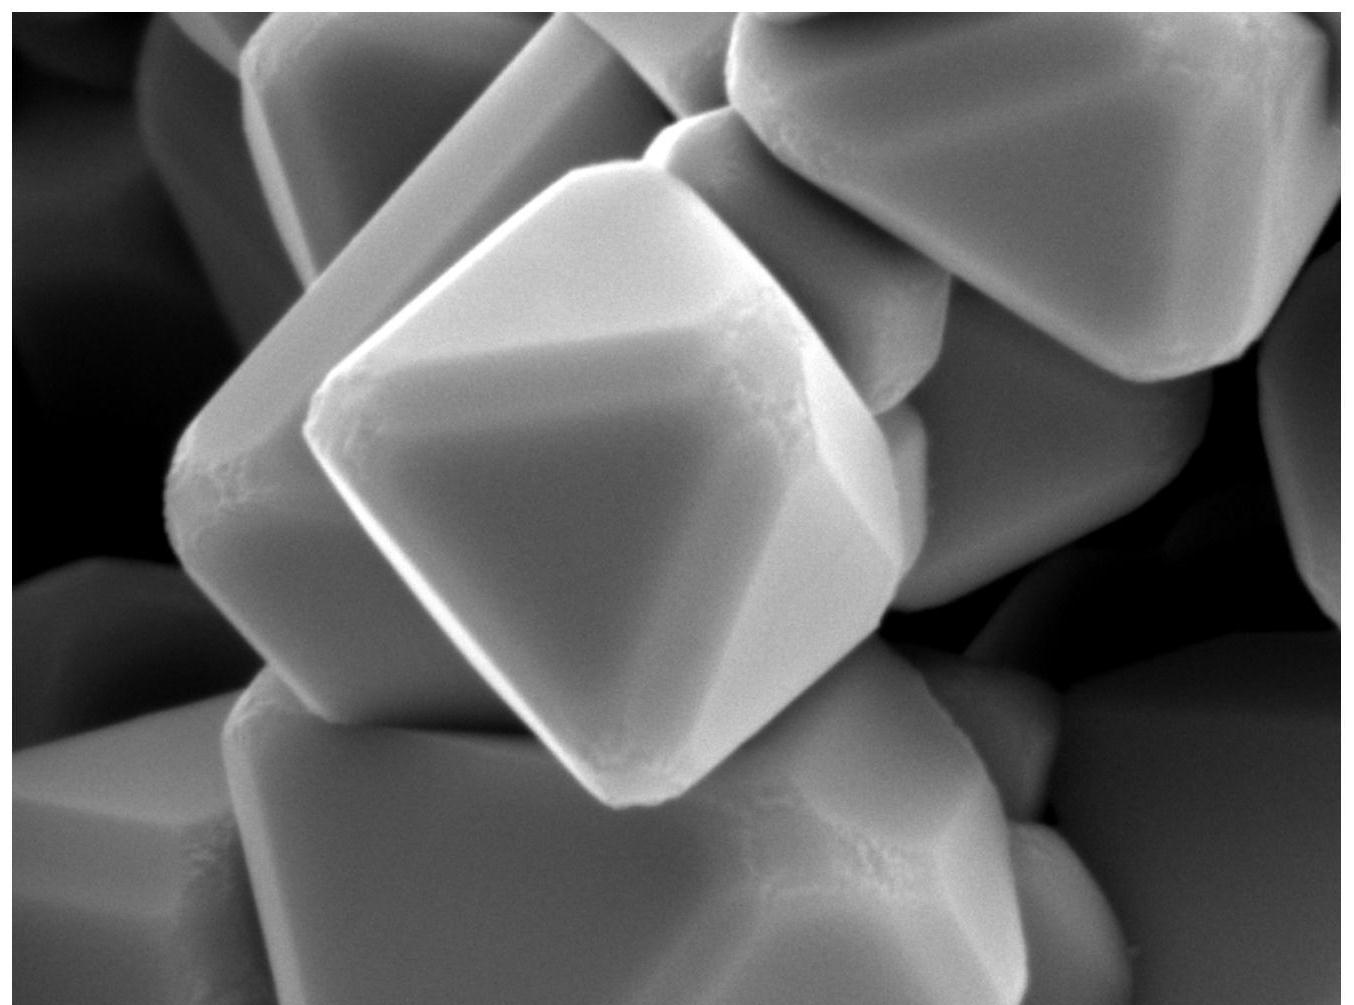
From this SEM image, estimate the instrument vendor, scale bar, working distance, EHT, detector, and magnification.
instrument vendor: JEOL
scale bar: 100 nm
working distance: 9.6 mm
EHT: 5 kV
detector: LED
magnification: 50 K X